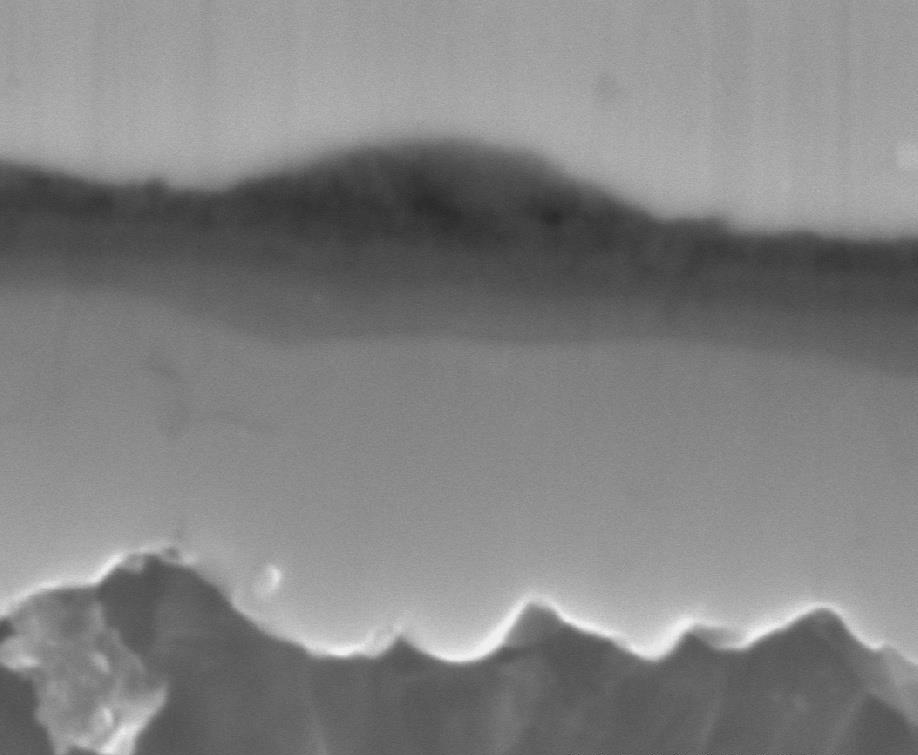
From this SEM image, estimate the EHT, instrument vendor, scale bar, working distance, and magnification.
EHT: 25 kV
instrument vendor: Hitachi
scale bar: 2000 nm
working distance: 7.5 mm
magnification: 15 K X